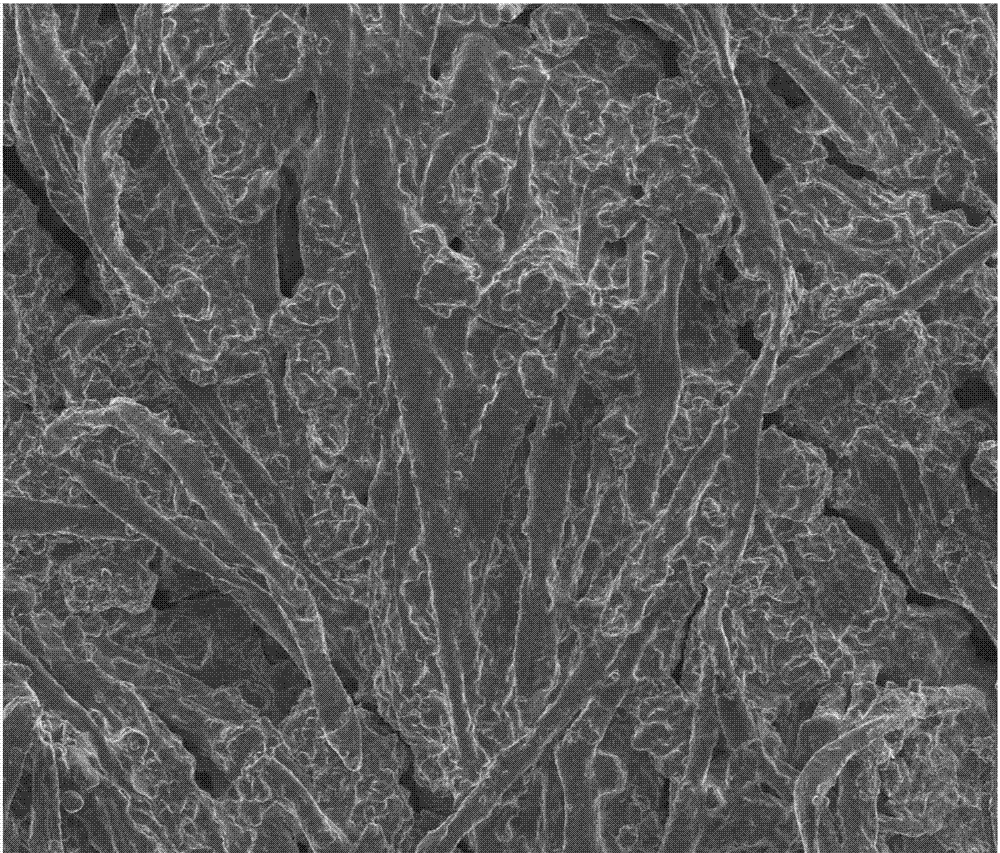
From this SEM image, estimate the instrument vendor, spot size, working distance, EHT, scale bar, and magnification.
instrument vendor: FEI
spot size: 3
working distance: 18.6 mm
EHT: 5 kV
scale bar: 300000 nm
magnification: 0.4 K X